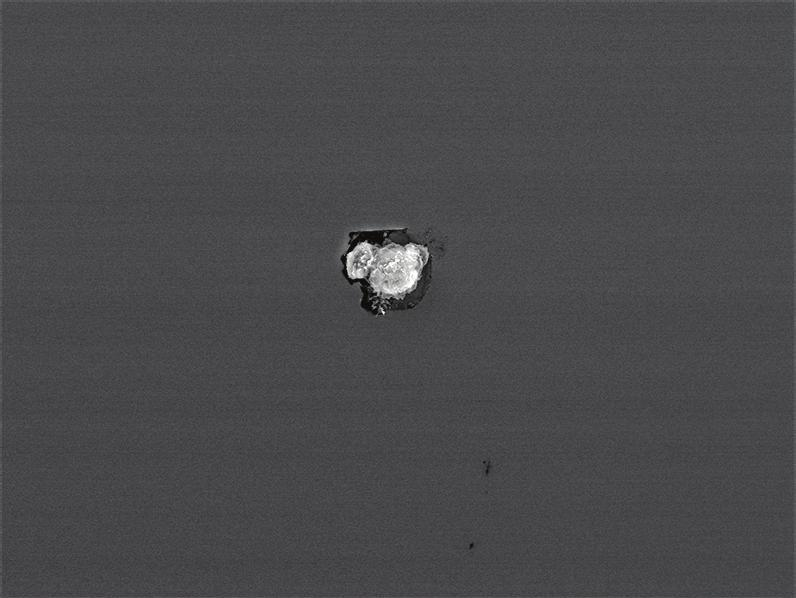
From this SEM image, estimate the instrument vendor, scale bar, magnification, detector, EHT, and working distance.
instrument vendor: JEOL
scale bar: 10000 nm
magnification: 0.85 K X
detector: SEI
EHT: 15 kV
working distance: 14.6 mm